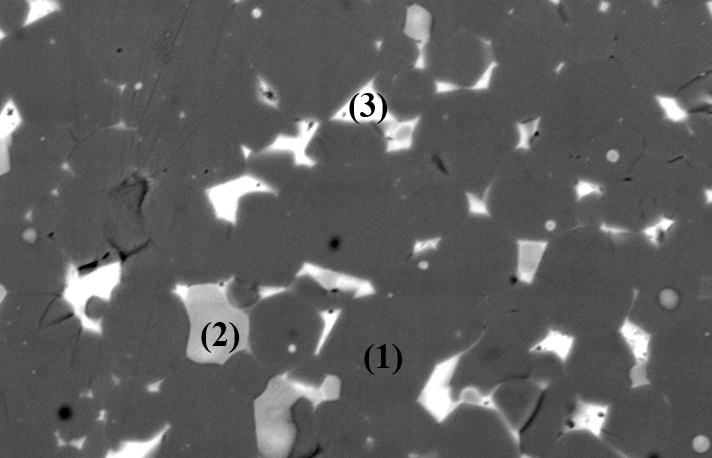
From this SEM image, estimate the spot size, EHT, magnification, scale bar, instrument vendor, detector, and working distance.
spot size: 4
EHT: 20 kV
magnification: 1.6 K X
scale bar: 10000 nm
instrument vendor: FEI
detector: BSE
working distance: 10 mm